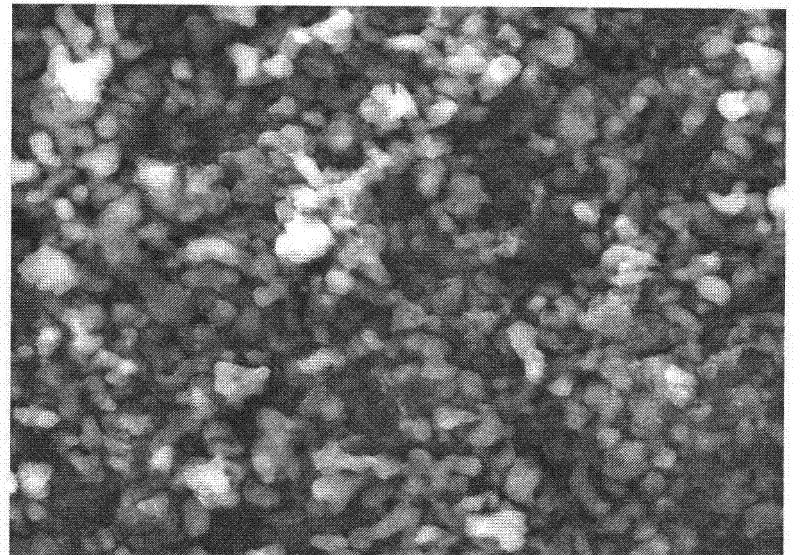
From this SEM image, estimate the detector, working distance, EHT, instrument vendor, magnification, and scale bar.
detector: ETD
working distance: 7.7 mm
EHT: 30 kV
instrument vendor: FEI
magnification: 6 K X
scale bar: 10000 nm